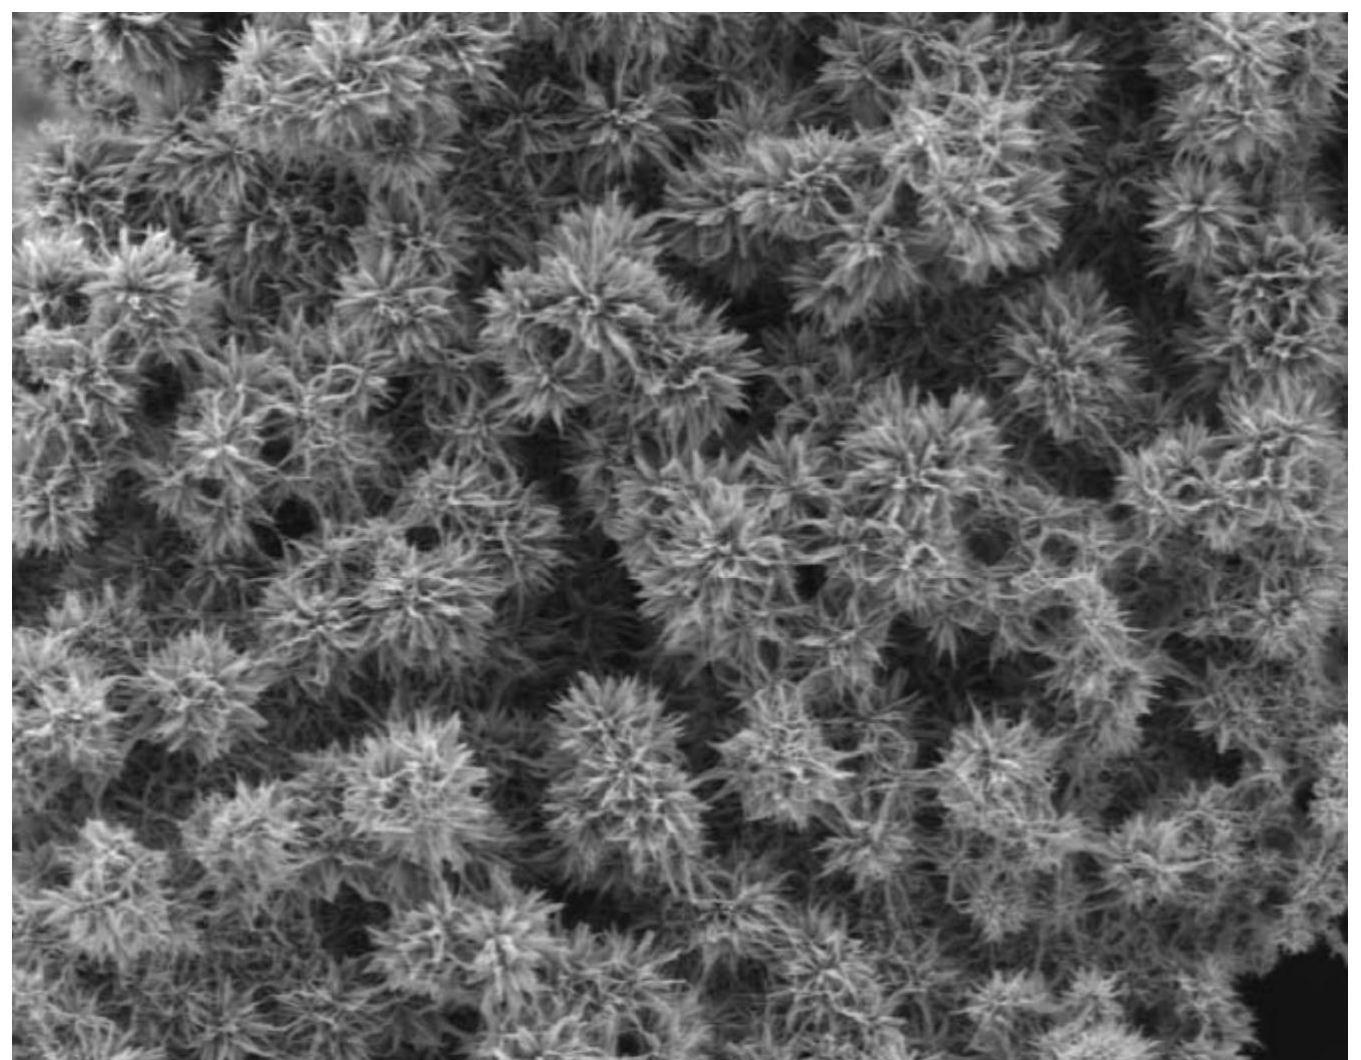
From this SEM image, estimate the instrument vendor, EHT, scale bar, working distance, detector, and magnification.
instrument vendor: JEOL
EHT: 3 kV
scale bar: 10000 nm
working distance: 7.8 mm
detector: LED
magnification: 2 K X